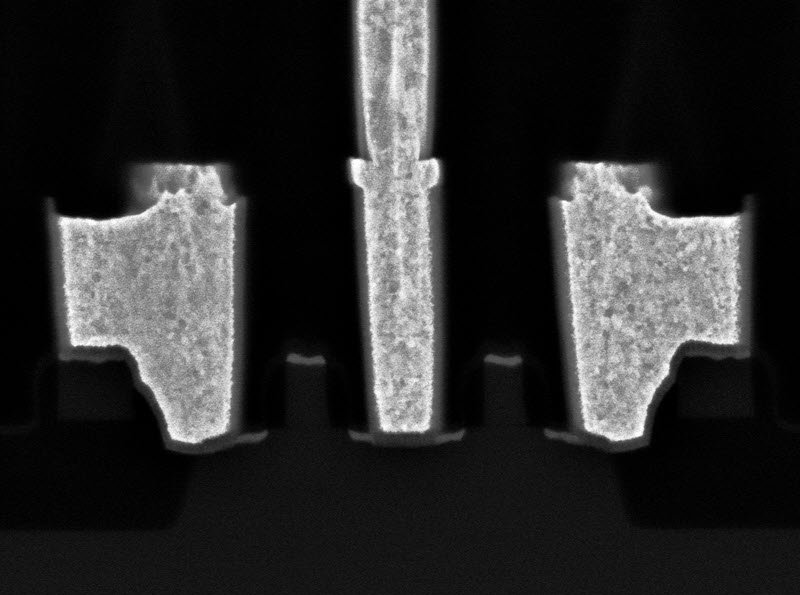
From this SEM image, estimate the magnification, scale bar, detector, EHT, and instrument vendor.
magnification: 50 K X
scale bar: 500 nm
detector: BED-C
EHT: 5 kV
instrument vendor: JEOL